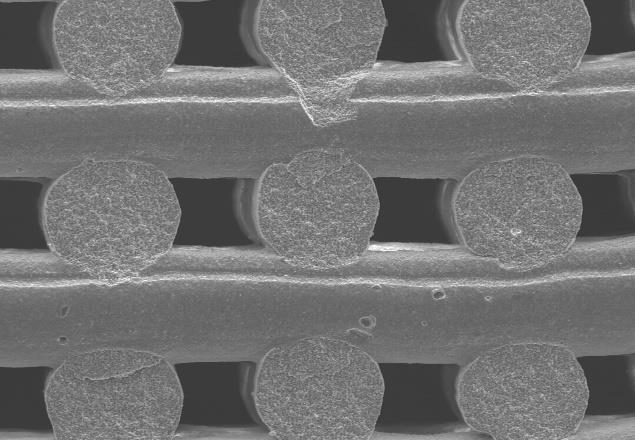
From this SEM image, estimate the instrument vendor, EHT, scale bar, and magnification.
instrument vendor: JEOL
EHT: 25 kV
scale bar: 500000 nm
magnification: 0.06 K X